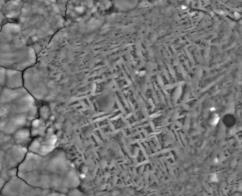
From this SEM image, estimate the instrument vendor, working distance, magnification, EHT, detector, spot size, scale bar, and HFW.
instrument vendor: FEI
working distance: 5.4 mm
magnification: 20 K X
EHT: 10 kV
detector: LVD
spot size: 4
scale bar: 5000 nm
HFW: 14.9 µm